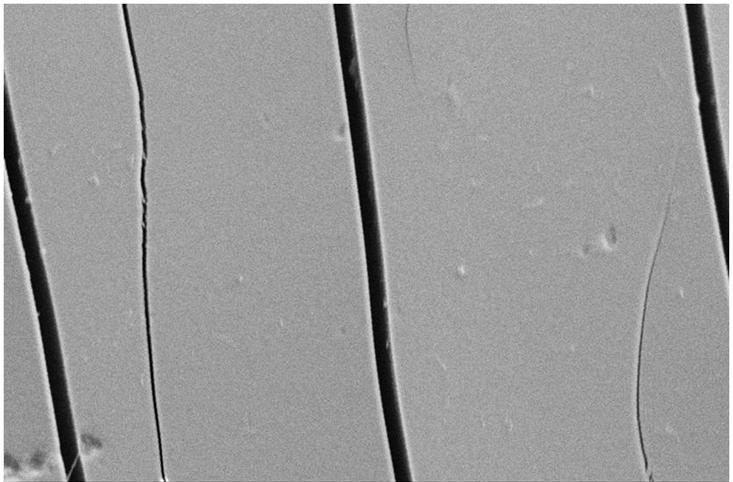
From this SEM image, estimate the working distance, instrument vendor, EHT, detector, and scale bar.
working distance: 5 mm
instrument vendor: Zeiss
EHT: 10 kV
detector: SE2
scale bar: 10000 nm